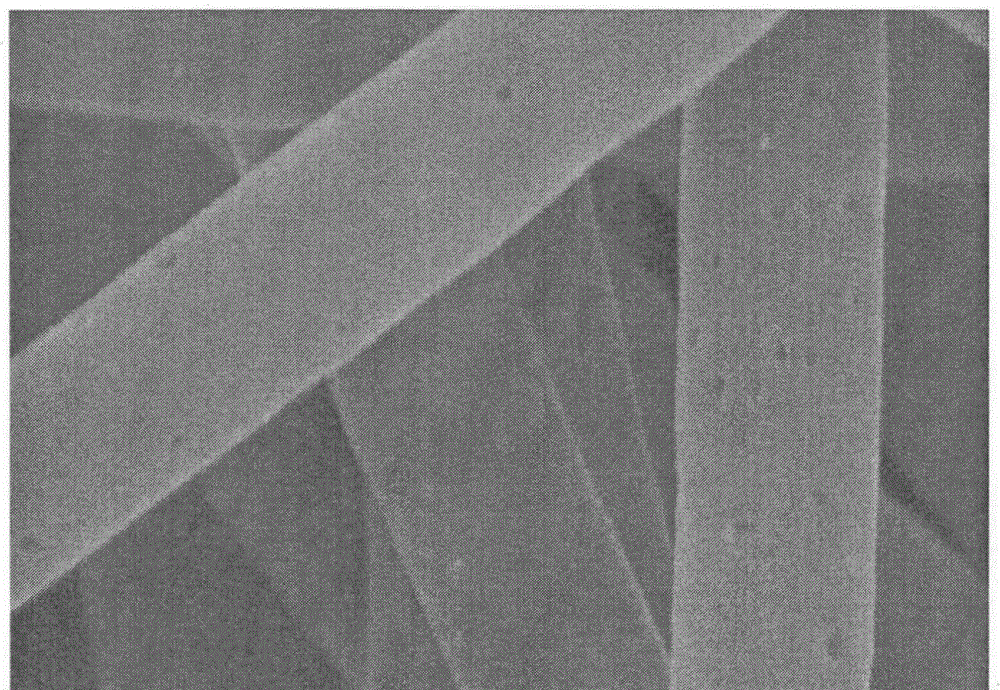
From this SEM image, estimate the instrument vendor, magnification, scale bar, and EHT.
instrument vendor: Hitachi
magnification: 100 K X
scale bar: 500 nm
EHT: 5 kV